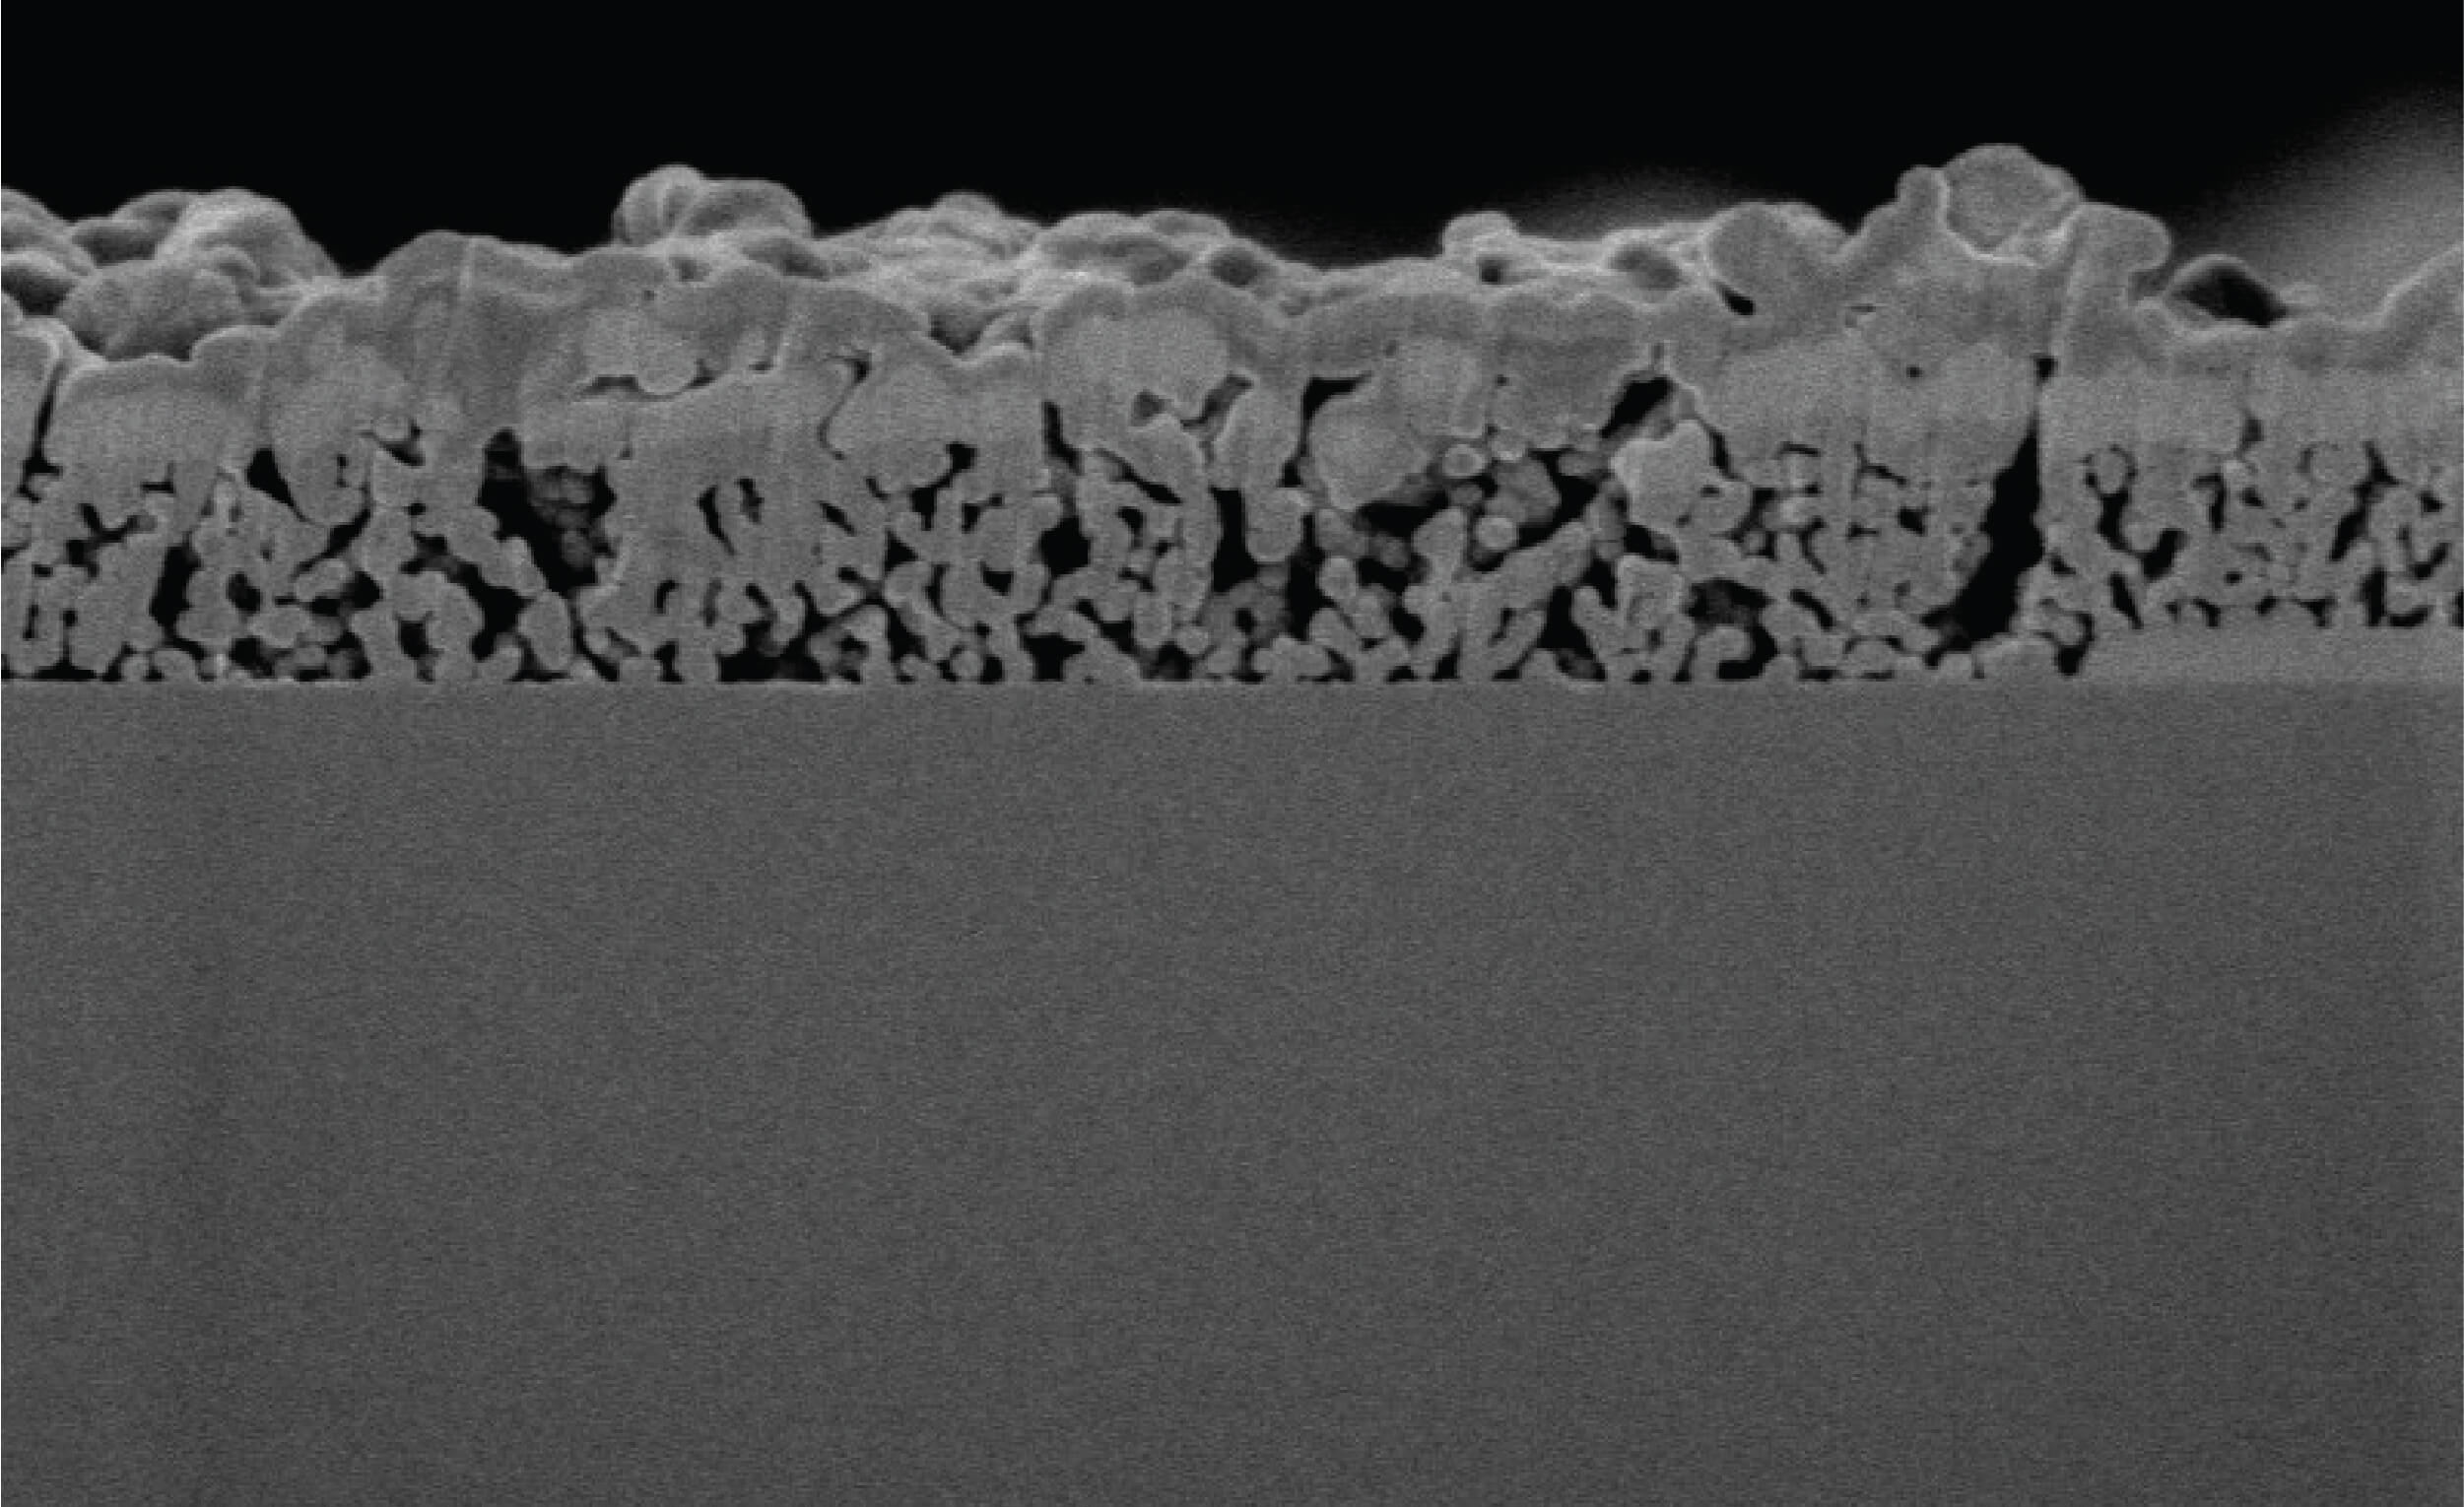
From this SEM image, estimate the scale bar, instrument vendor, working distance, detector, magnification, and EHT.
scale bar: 2000 nm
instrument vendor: Hitachi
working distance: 4 mm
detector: SE(UL)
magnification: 25 K X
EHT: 1 kV